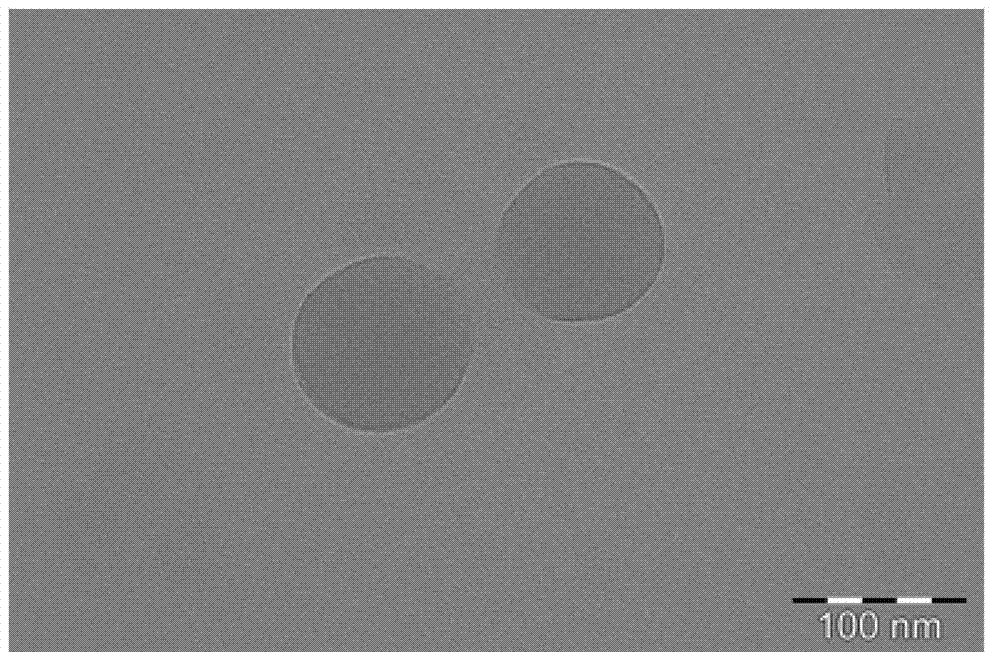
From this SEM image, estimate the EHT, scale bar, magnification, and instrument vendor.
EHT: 200 kV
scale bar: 100 nm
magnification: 97 K X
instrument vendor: FEI